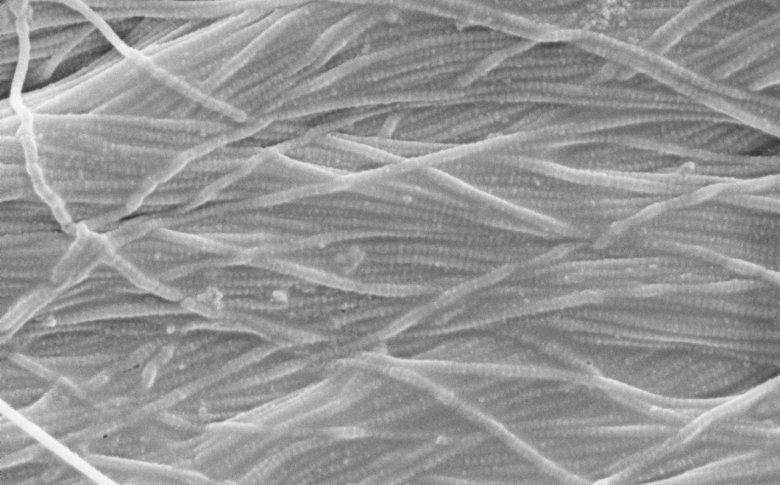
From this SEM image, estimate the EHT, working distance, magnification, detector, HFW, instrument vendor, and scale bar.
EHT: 5 kV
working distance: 11.266 mm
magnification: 20 K X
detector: SED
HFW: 6.35 µm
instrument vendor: Thermo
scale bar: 2000 nm